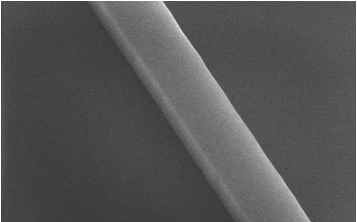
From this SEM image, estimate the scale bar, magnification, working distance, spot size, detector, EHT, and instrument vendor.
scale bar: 500 nm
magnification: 125 K X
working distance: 5 mm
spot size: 2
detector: TLD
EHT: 10 kV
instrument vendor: FEI